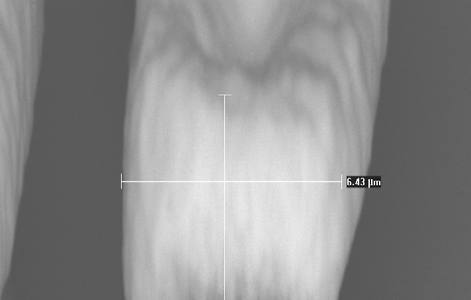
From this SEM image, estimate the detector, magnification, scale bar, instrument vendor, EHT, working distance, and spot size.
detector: BSE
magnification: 9.01 K X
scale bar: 2000 nm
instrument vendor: FEI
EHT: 30 kV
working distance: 10.5 mm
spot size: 2.5